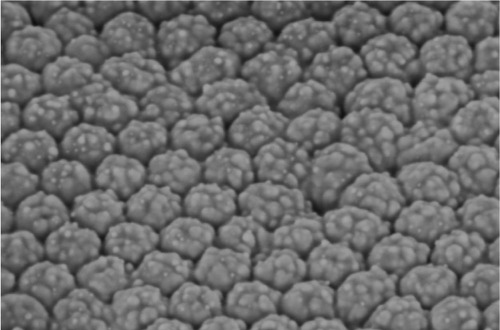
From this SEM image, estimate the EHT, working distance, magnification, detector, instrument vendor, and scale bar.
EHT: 2.34 kV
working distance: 5.8 mm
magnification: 100 K X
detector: InLens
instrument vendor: Zeiss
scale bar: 100 nm